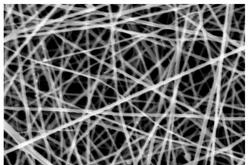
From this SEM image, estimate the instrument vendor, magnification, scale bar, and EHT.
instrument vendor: other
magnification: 5 K X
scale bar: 10000 nm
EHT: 20 kV